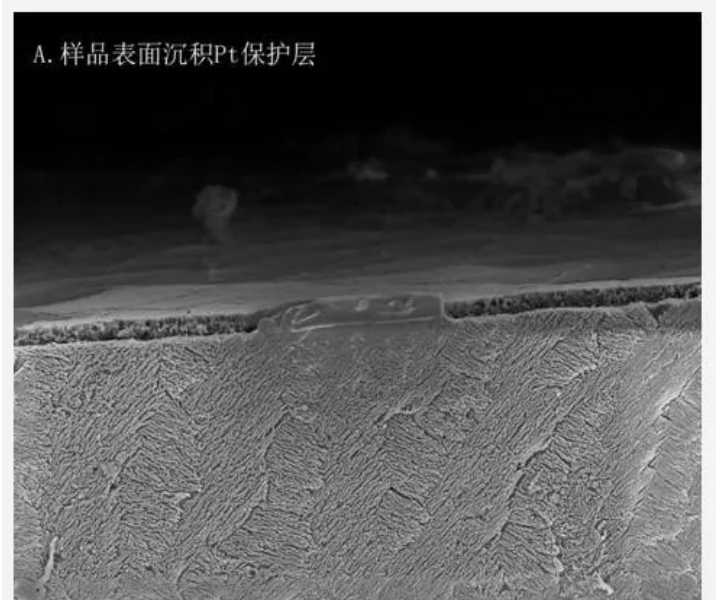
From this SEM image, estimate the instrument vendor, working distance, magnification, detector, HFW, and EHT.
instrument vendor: FEI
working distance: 4 mm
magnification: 7 K X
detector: ETD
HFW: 36.6 µm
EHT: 5 kV